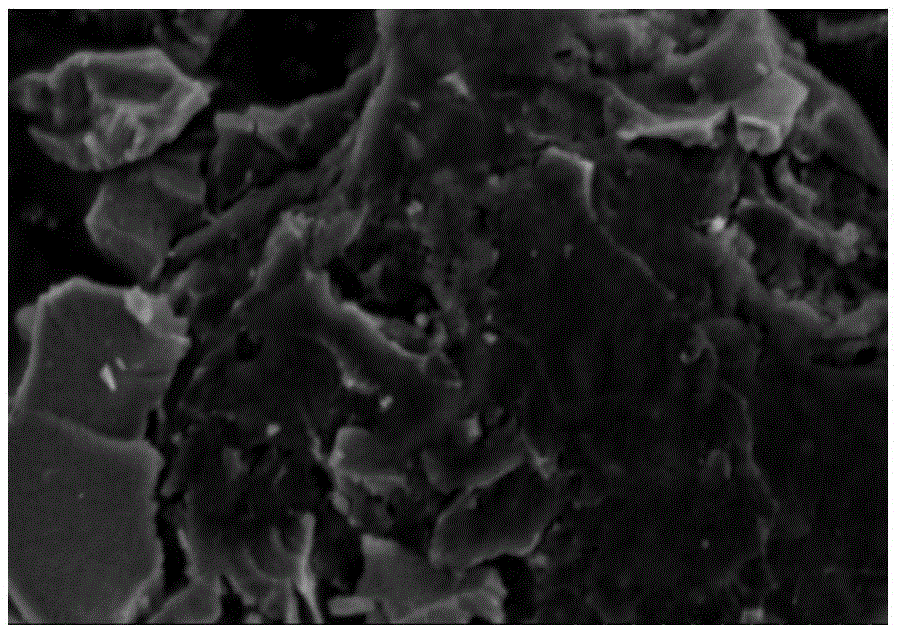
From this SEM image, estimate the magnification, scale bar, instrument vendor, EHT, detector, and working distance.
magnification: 8.5 K X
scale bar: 5000 nm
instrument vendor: Hitachi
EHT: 5 kV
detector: SE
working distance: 5.6 mm